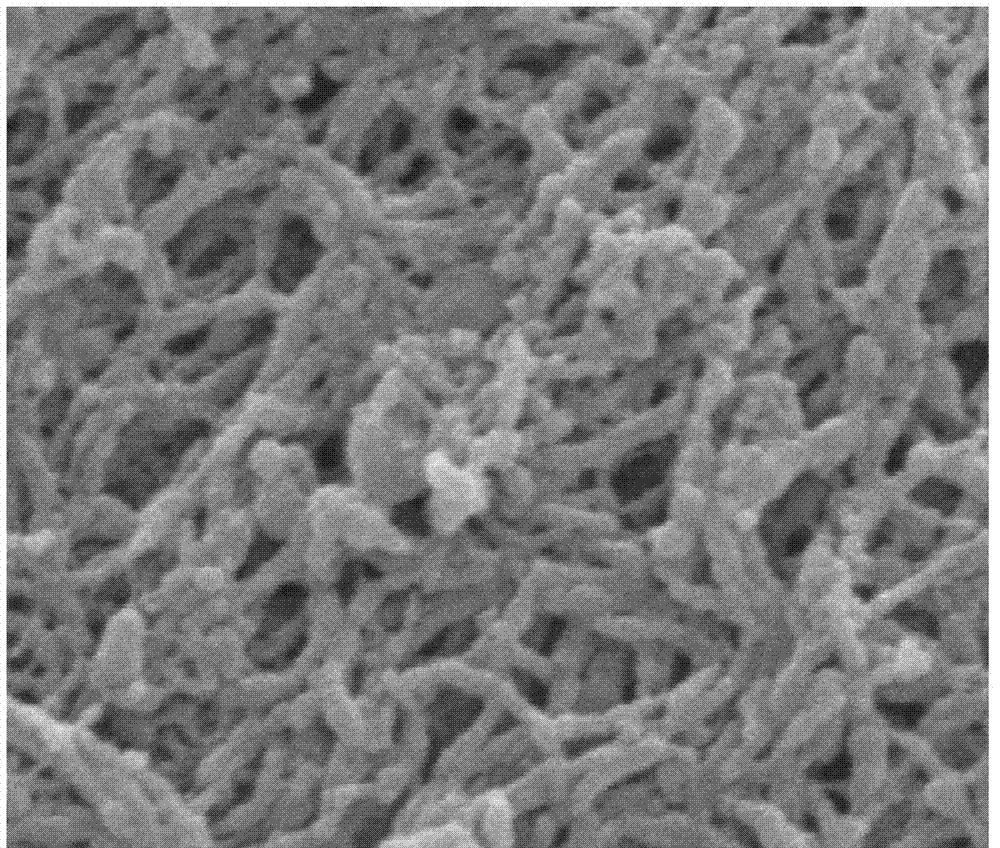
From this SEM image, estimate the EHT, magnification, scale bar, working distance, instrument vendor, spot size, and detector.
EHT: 15 kV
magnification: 30 K X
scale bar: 1000 nm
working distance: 9.4 mm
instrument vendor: FEI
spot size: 2.5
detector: ETD